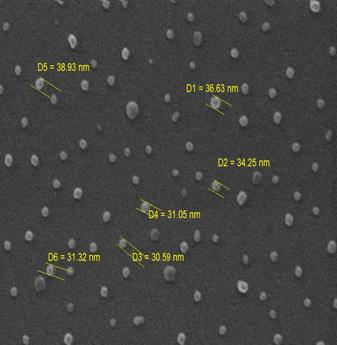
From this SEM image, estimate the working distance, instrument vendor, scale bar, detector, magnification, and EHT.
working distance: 4.832 mm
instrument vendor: Tescan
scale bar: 200 nm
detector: InBeam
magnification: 200 K X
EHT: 20 kV